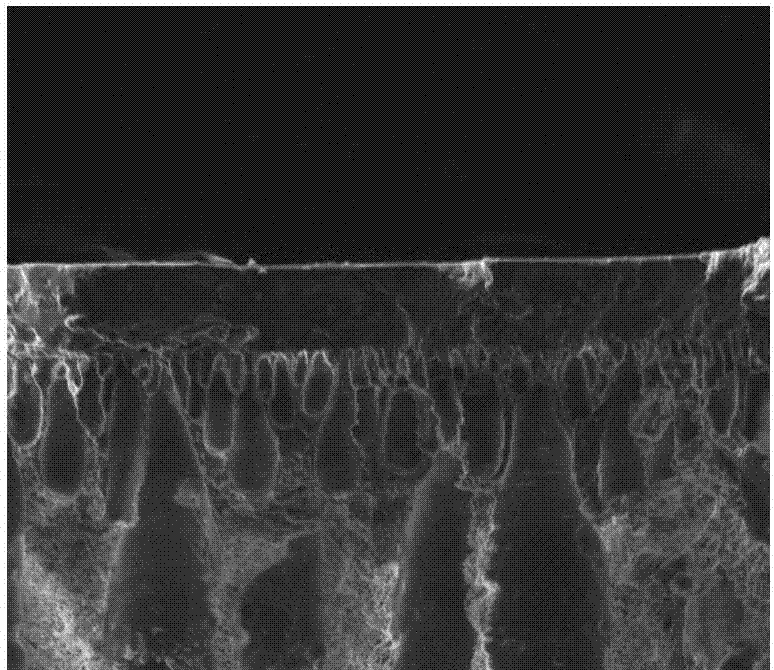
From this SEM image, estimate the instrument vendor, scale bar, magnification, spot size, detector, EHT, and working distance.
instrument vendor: FEI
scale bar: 30000 nm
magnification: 2 K X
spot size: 3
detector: ETD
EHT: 10 kV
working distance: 4.8 mm